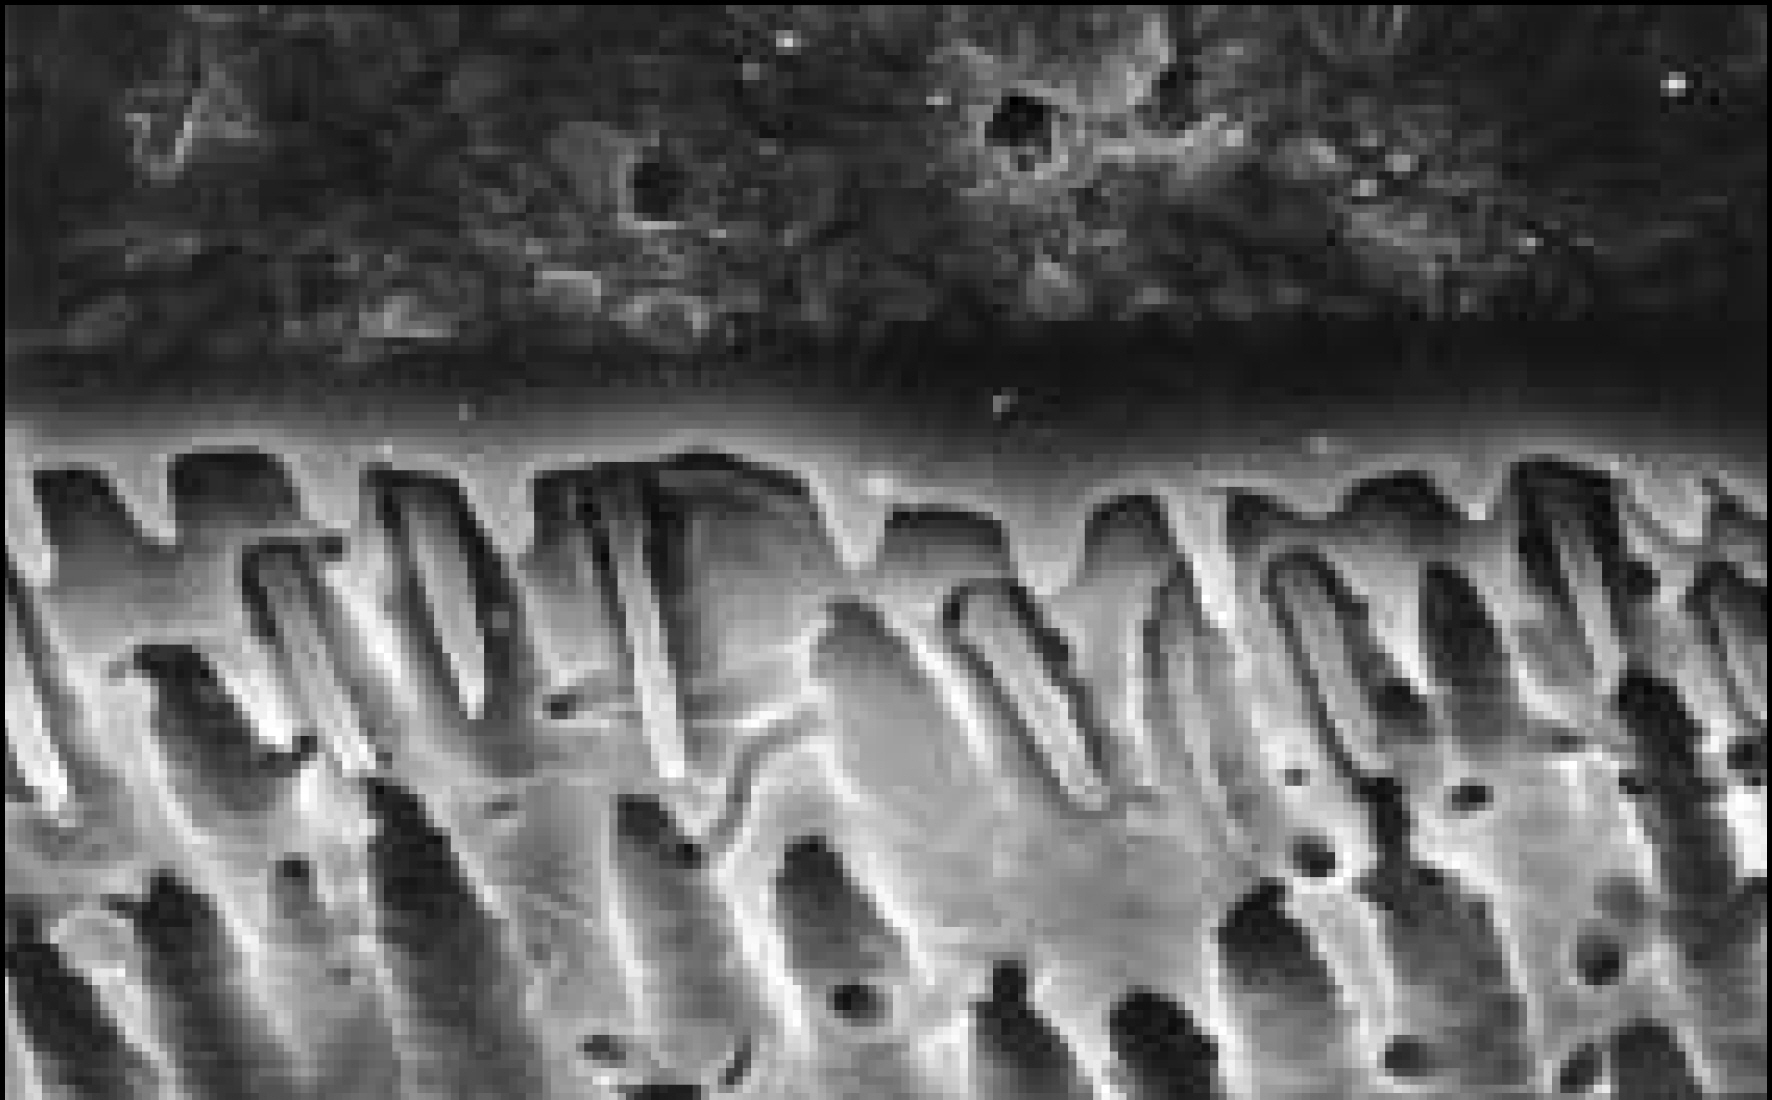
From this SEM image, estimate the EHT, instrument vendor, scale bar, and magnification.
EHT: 20 kV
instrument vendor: Hitachi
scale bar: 20000 nm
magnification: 2 K X